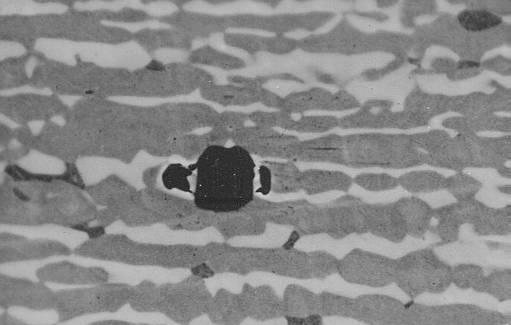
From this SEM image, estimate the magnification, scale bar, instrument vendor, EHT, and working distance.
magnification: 2.2 K X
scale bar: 10000 nm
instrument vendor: JEOL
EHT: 20 kV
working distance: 14 mm